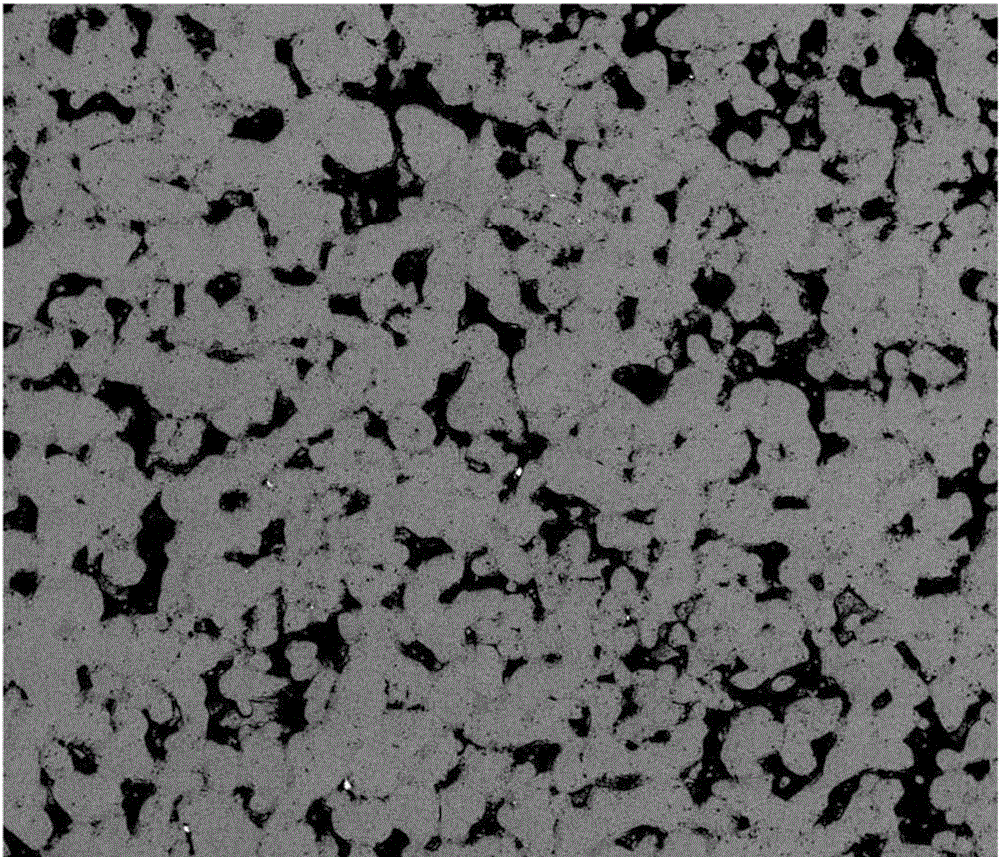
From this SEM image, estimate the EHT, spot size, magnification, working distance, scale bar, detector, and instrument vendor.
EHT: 20 kV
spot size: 7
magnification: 0.2 K X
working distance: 10 mm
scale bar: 500000 nm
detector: DualBSD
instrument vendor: FEI